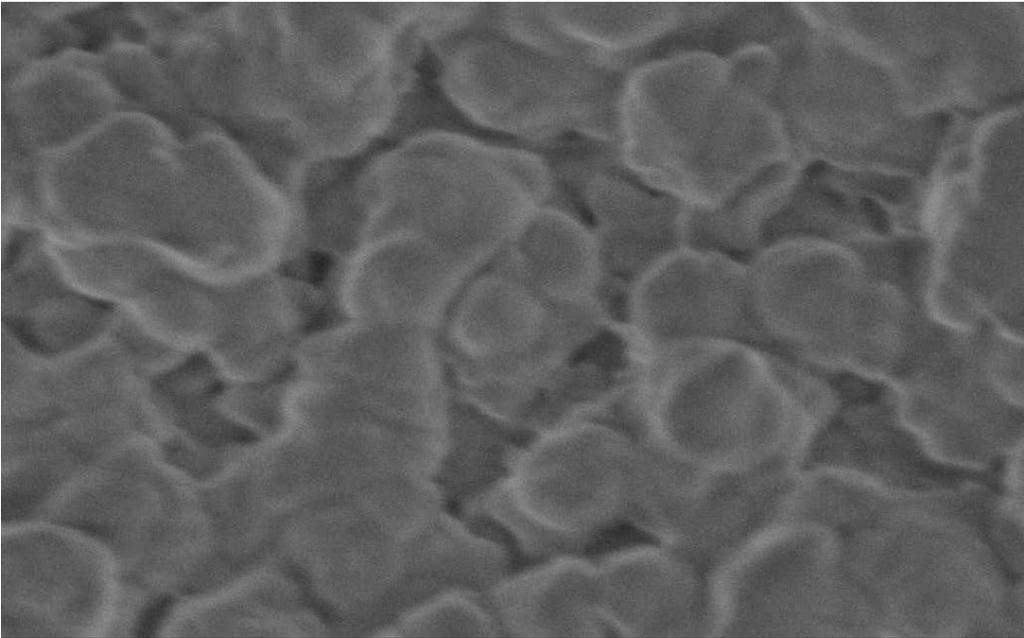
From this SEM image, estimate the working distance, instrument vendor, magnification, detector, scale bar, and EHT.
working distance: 2.4 mm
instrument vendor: Hitachi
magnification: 100 K X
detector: SE(U,LA2)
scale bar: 500 nm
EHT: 0.5 kV